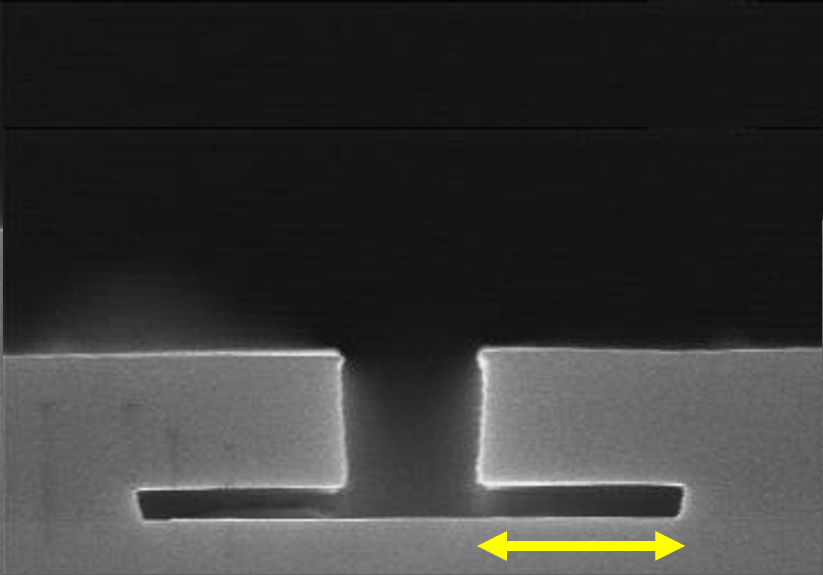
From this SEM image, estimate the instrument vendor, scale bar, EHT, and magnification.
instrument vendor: JEOL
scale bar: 1200 nm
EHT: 15 kV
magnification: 25 K X